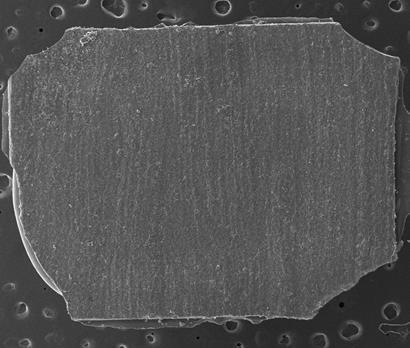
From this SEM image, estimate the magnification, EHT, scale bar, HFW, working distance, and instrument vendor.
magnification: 0.07 K X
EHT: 20 kV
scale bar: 2000000 nm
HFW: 4260 µm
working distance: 30.1 mm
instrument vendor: FEI